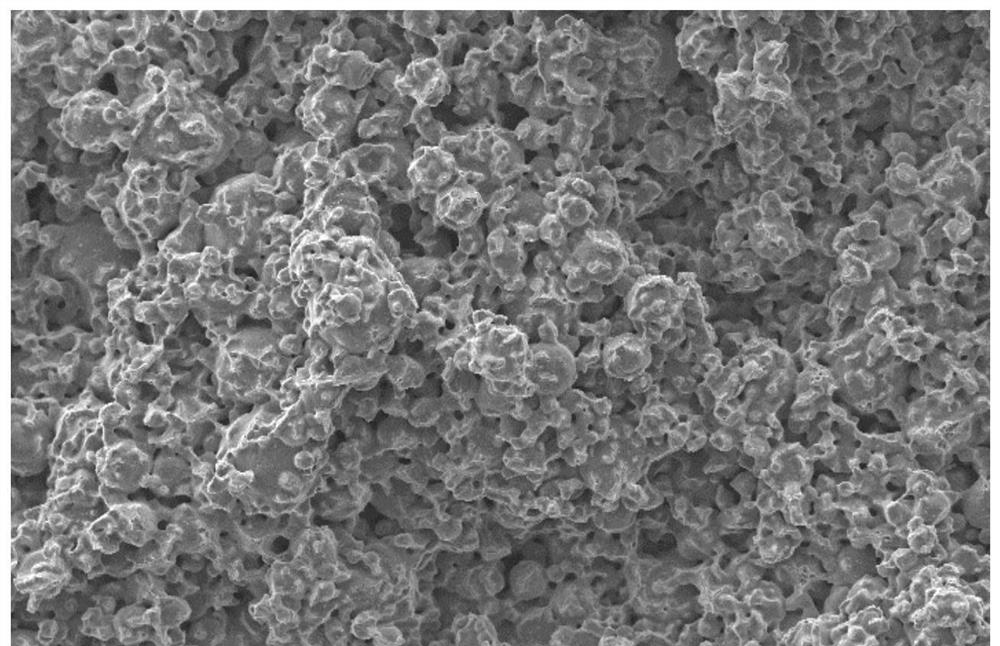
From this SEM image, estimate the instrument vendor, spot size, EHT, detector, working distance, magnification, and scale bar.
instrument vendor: FEI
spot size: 3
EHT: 15 kV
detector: SE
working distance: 5.9 mm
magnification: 0.5 K X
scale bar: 50000 nm